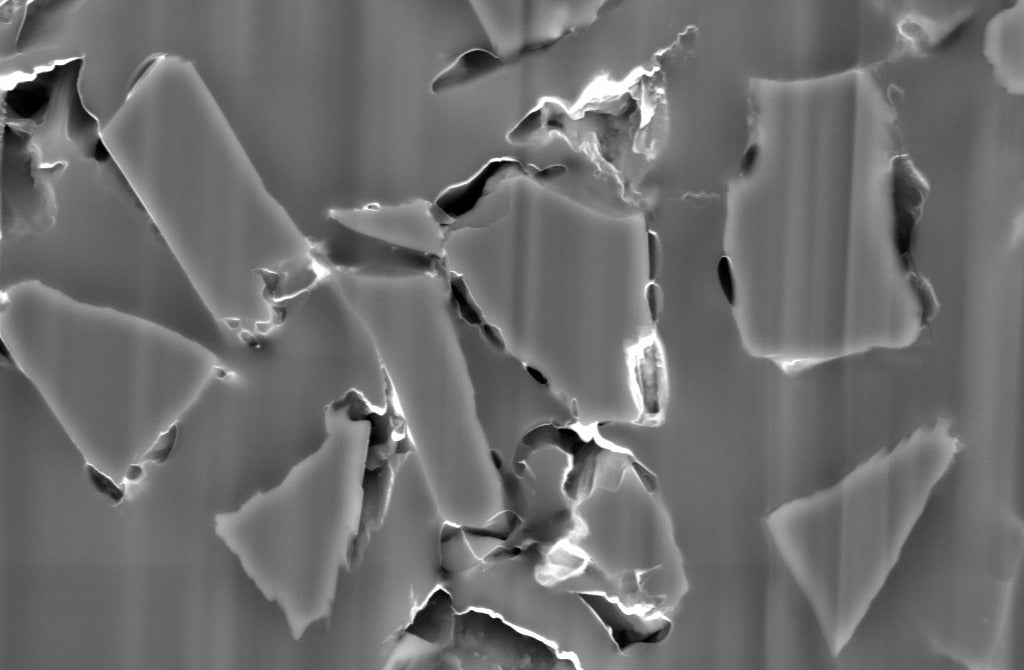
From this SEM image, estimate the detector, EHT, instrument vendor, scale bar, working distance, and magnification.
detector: BSD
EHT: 10 kV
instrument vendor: Zeiss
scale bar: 1000 nm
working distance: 10.9 mm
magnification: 5 K X